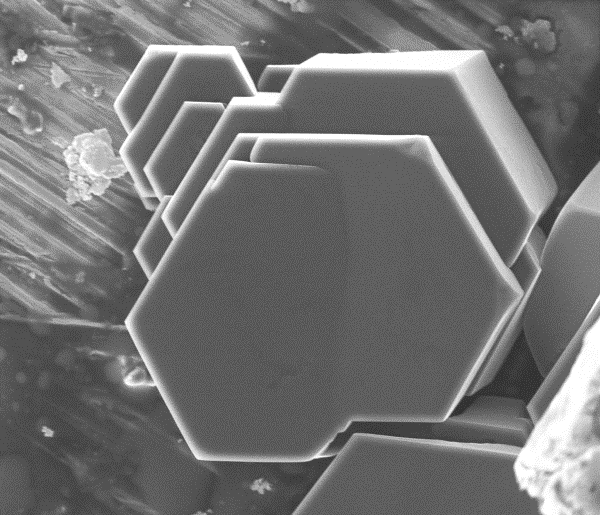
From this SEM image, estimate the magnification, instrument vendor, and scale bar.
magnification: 0.987 K X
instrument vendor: FEI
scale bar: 50000 nm